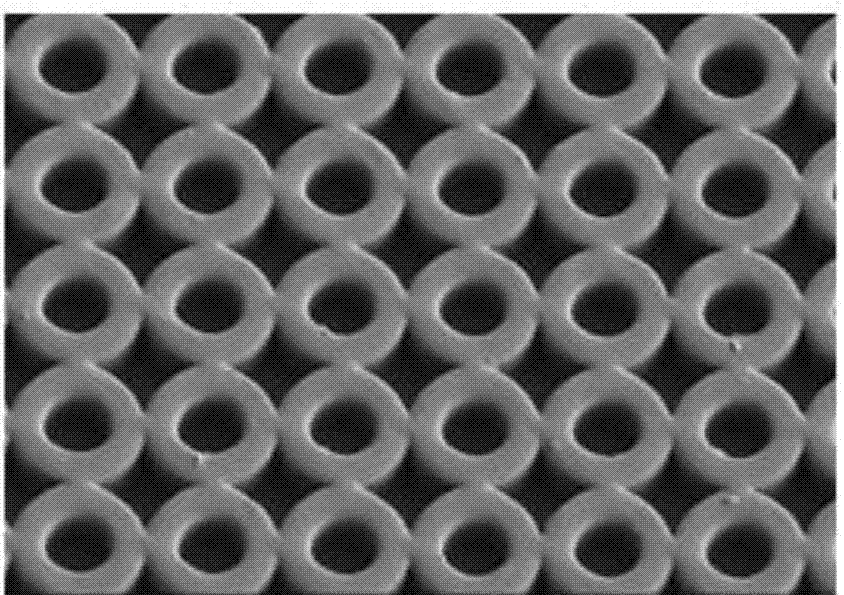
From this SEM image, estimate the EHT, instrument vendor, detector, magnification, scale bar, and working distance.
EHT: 1 kV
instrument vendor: Hitachi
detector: SE(L)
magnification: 1 K X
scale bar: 50000 nm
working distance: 8.8 mm